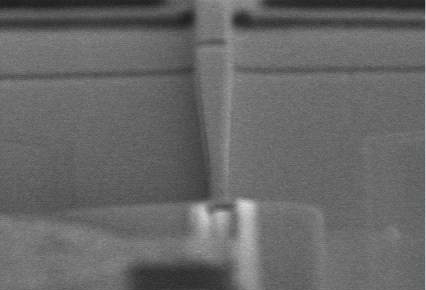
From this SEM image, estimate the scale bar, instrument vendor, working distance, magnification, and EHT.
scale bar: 10000 nm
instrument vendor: Hitachi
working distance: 11.1 mm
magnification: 3 K X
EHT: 10 kV